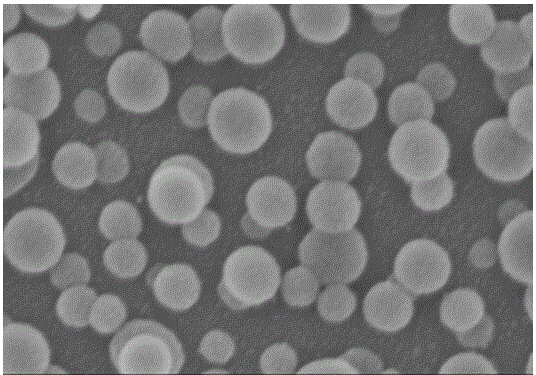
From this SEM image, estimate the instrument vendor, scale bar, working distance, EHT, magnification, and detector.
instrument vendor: Hitachi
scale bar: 500 nm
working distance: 9.1 mm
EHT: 3 kV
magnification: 100 K X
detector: SE(M)